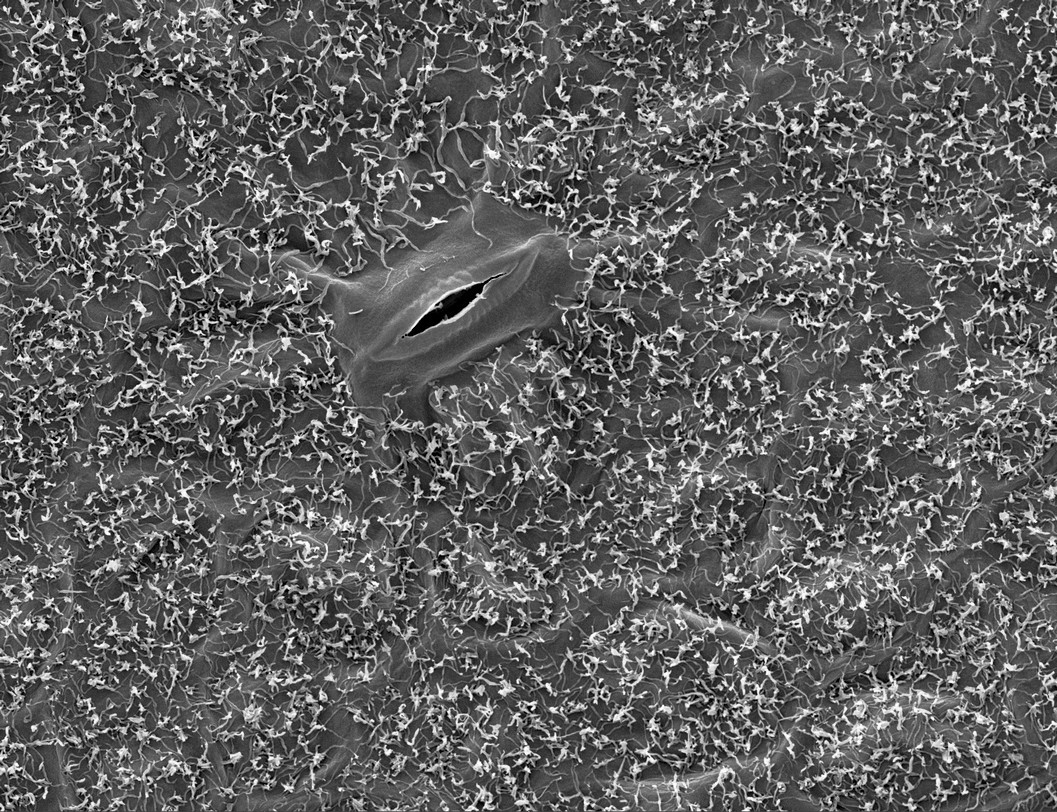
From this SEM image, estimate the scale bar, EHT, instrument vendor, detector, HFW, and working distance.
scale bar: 20000 nm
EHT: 10 kV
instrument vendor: Tescan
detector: SE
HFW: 105 µm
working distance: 8 mm